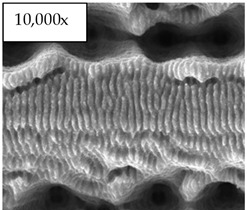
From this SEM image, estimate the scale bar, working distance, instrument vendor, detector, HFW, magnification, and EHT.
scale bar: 10000 nm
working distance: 7 mm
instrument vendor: FEI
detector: ETD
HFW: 29.8 µm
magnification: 10 K X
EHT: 30 kV